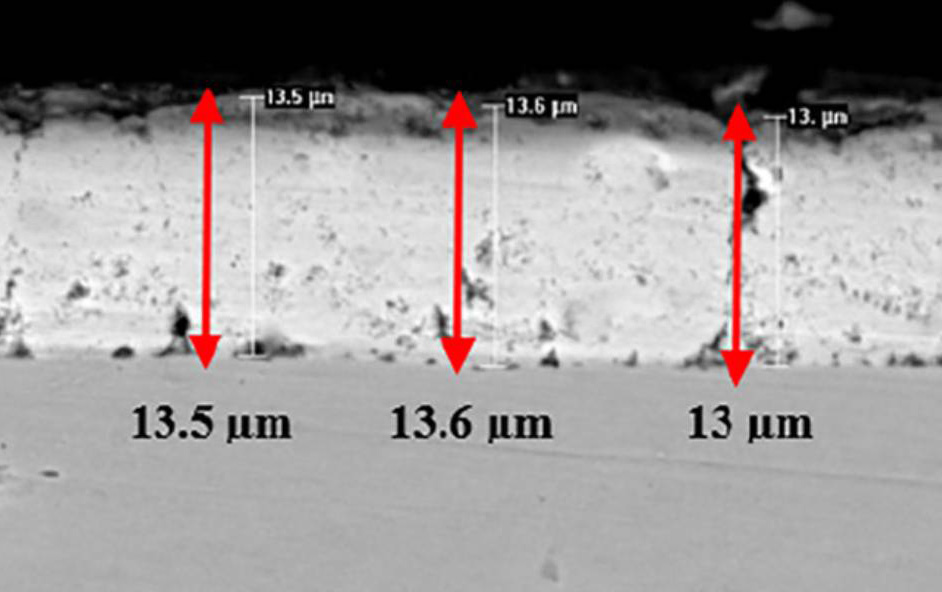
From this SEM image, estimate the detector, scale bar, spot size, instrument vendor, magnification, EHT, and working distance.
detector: BSE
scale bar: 10000 nm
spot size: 5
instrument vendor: FEI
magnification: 5 K X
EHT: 20 kV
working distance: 11.7 mm